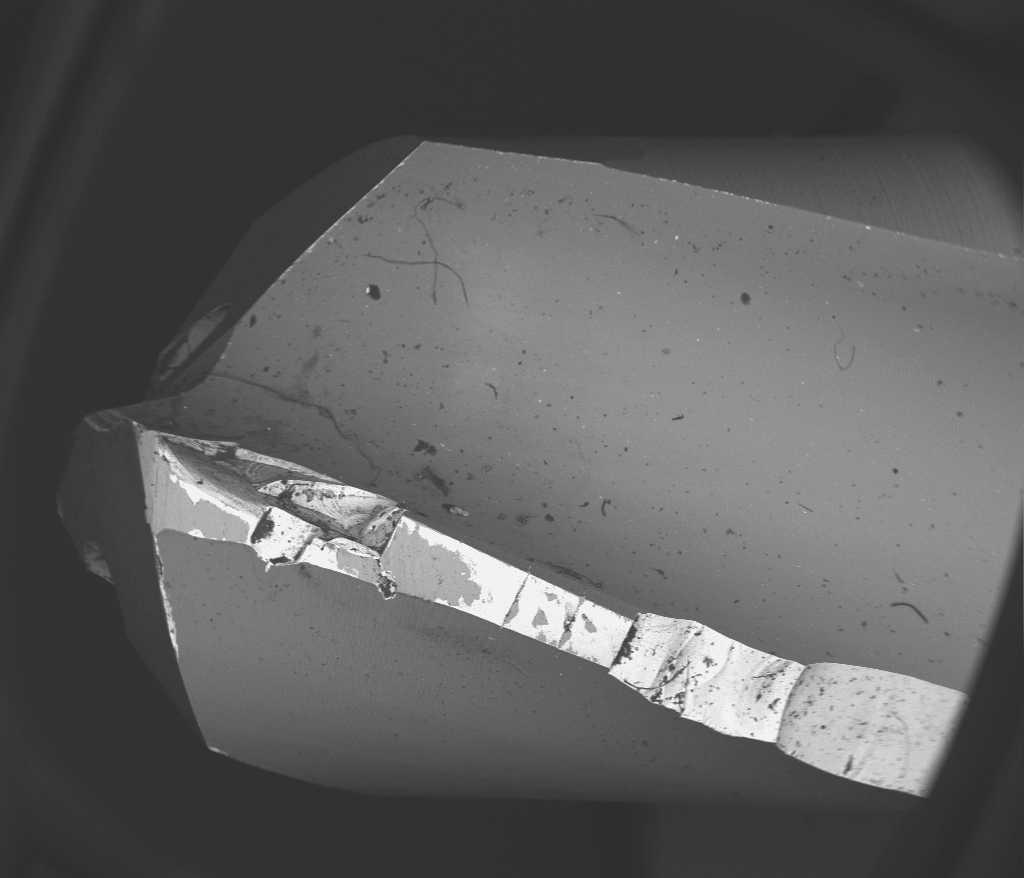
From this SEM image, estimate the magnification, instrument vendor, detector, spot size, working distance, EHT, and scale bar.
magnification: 0.014 K X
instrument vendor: FEI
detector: SSD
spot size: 4.5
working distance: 18 mm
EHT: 30 kV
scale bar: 5000000 nm